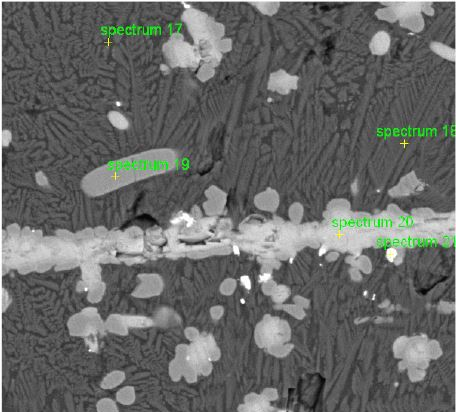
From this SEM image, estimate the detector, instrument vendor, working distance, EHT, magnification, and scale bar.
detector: BSE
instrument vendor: other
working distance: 15.4 mm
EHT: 15 kV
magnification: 3.638 K X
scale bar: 10000 nm